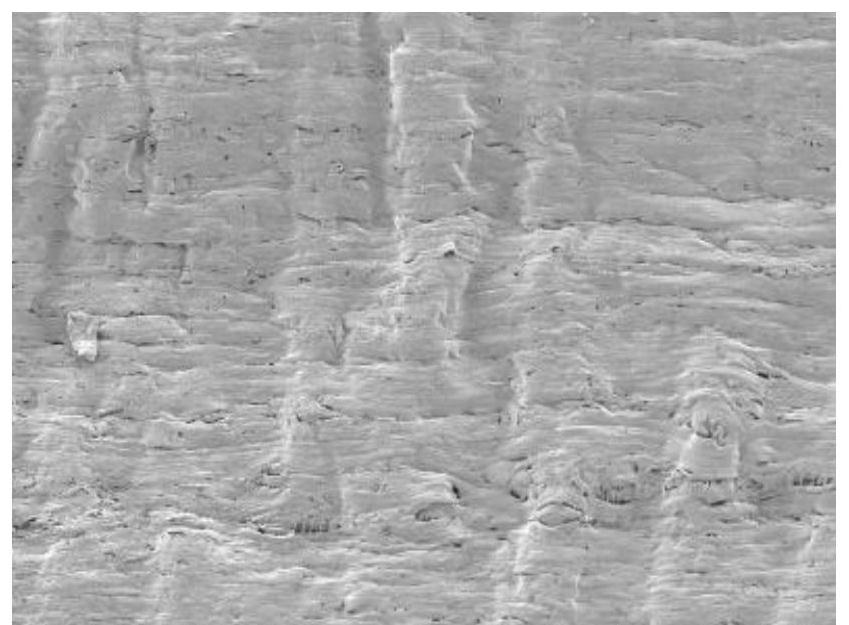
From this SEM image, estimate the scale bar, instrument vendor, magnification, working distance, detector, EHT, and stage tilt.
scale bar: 100000 nm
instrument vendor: Tescan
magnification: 1 K X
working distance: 7.56 mm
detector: SE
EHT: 2 kV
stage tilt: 0°